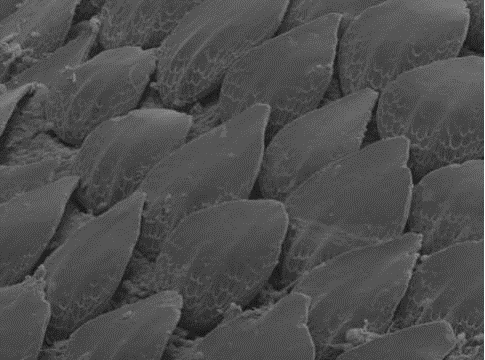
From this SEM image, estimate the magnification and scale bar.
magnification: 0.187 K X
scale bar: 20000 nm